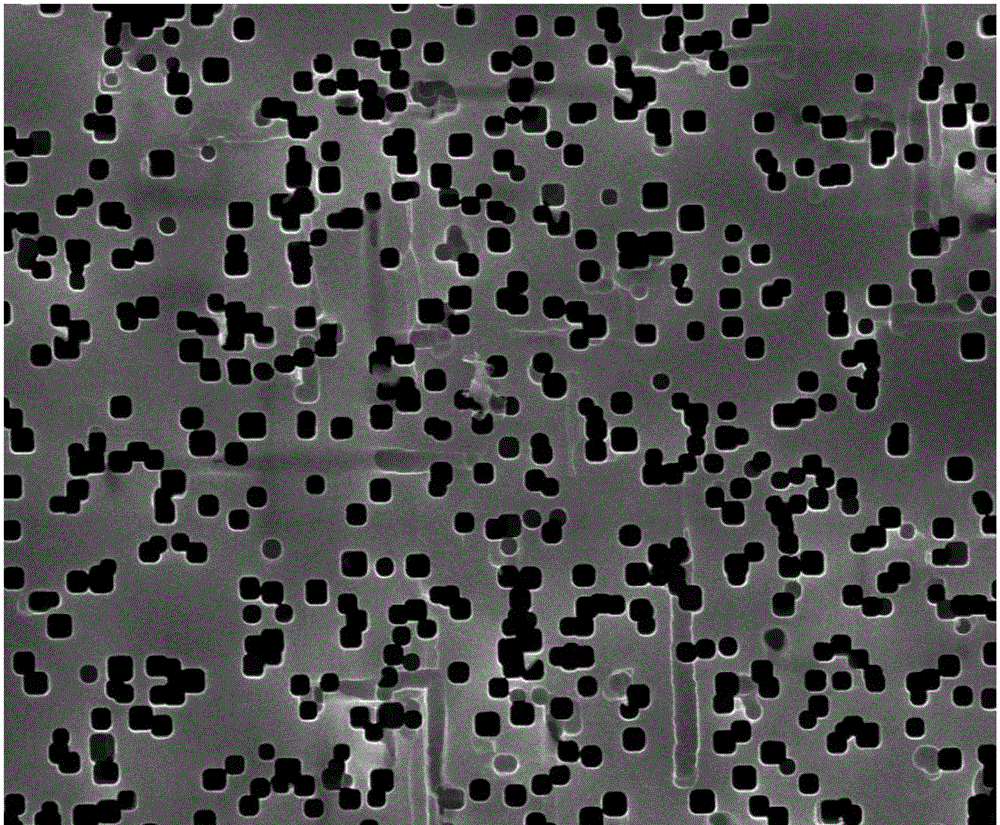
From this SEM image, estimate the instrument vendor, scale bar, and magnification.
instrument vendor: JEOL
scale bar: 10000 nm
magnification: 2 K X